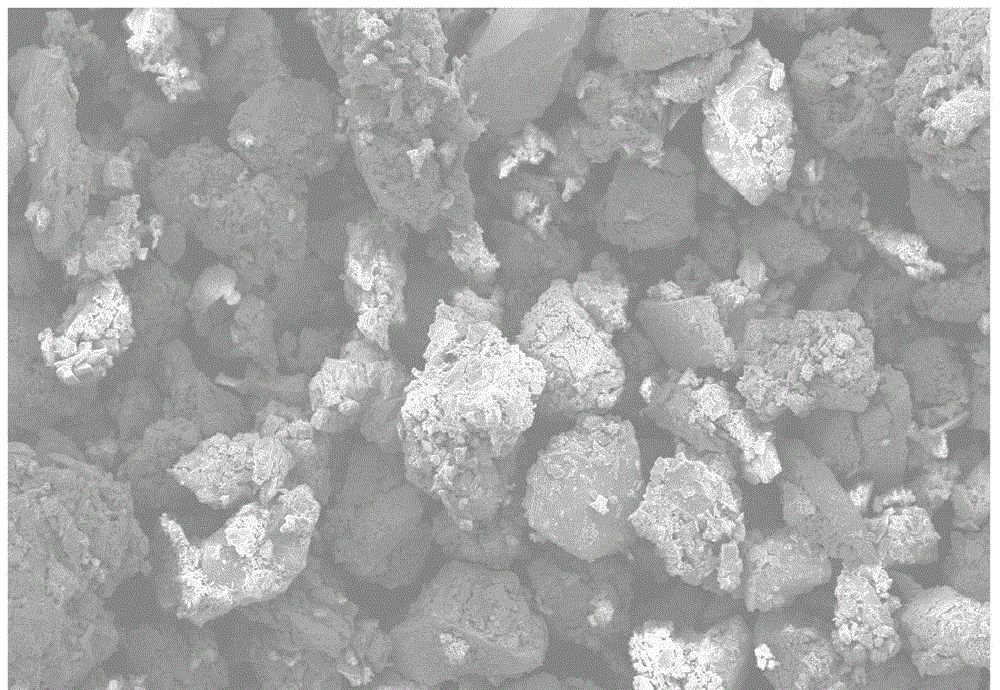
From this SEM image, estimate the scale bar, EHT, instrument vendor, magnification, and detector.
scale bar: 100000 nm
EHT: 20 kV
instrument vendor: JEOL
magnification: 0.1 K X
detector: SEI